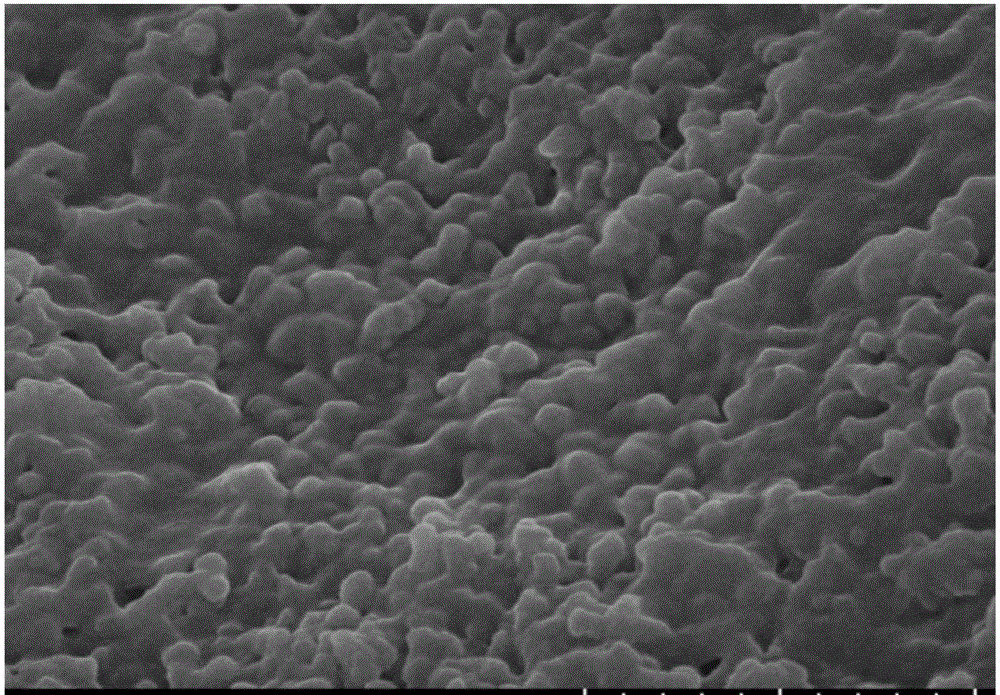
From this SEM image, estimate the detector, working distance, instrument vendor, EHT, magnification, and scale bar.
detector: SE(UL)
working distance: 10.9 mm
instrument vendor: Hitachi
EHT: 5 kV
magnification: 50 K X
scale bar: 1000 nm